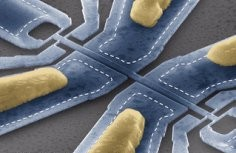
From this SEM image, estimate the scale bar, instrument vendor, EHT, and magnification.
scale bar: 2000 nm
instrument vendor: FEI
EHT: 15 kV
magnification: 10 K X